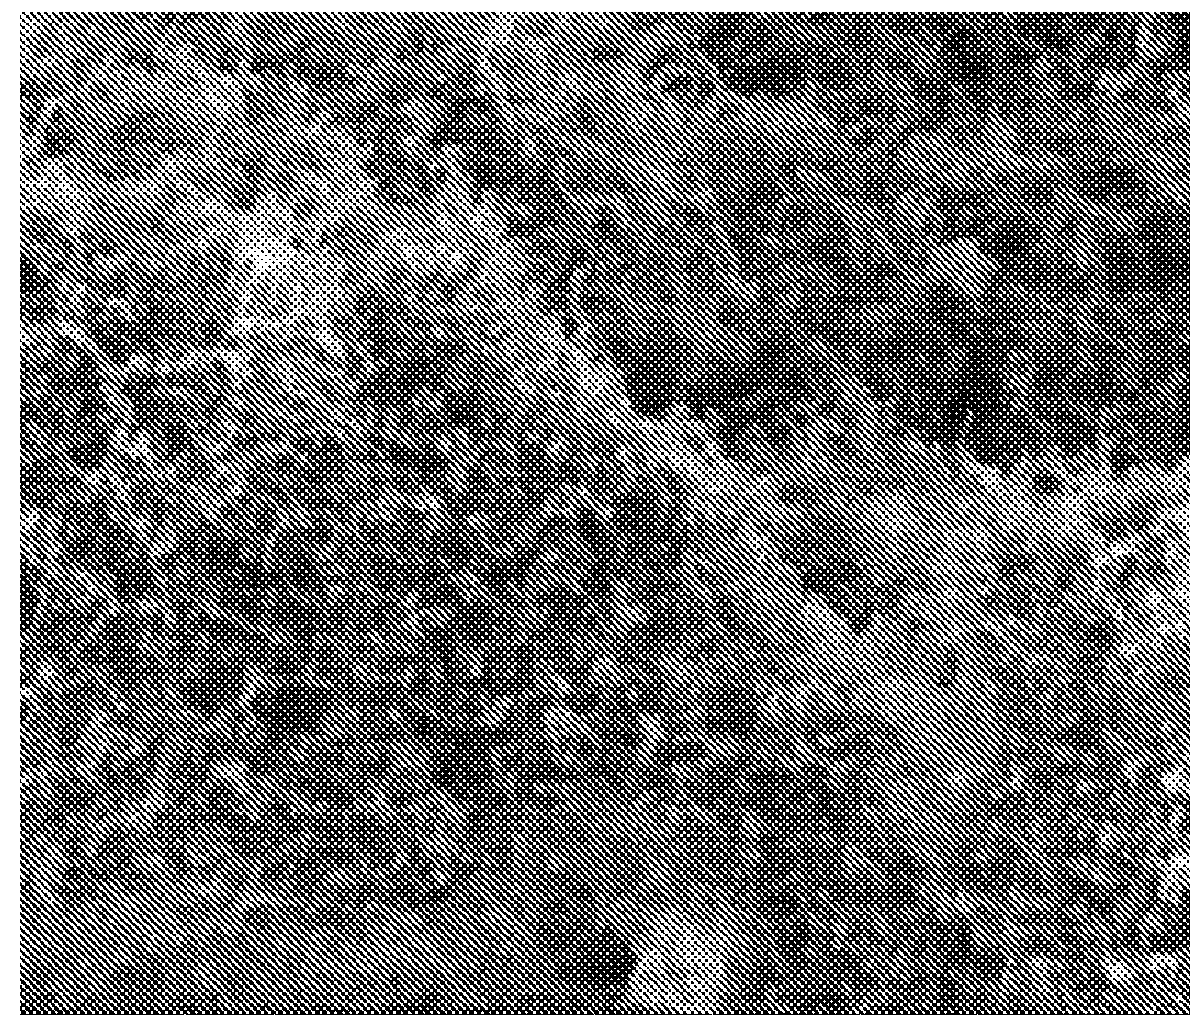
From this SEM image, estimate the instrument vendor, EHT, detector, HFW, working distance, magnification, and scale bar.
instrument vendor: FEI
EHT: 5 kV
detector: ETD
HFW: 30.6 µm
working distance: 5.1 mm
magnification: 10 K X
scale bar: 5000 nm